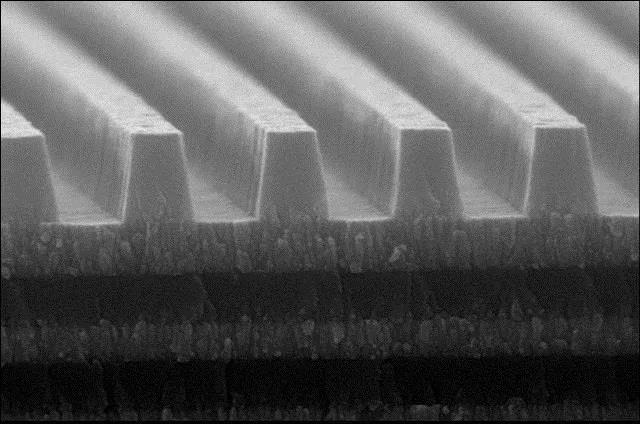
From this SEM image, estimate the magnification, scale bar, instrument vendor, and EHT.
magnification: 43.2 K X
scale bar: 1000 nm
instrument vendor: other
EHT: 15 kV